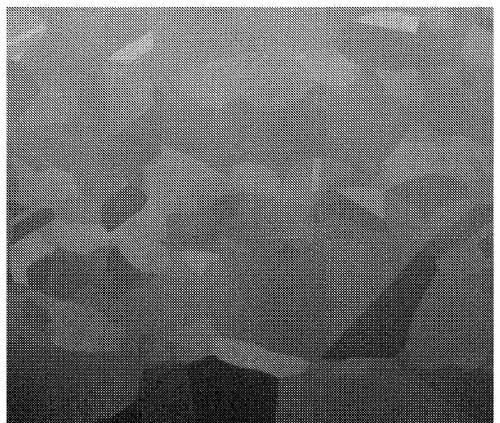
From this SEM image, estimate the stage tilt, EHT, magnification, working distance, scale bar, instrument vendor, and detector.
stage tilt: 52°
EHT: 2 kV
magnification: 10 K X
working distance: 10.1 mm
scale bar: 5000 nm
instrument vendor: FEI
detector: ETD